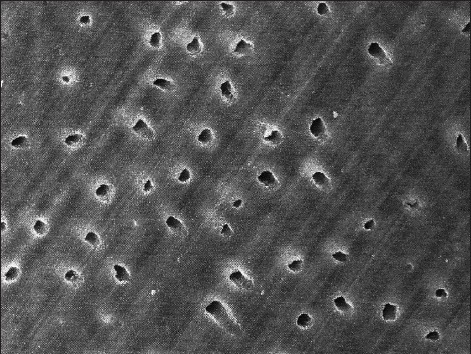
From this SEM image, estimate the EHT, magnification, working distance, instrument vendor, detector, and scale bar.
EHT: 15 kV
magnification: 3.2 K X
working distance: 33 mm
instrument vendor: Zeiss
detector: SE1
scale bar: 2000 nm